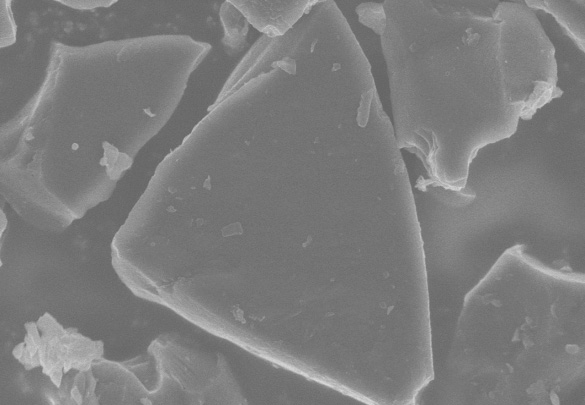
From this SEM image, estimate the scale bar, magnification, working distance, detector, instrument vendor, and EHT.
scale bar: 5000 nm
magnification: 10 K X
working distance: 8.4 mm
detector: SE(U)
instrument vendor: Hitachi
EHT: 5 kV